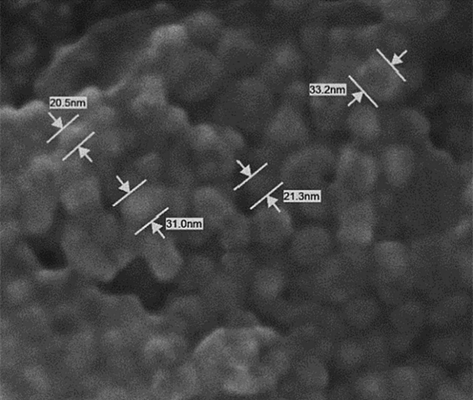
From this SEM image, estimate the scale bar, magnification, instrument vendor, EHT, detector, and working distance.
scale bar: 100 nm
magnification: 250 K X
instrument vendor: JEOL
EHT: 5 kV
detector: SEI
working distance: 7.5 mm